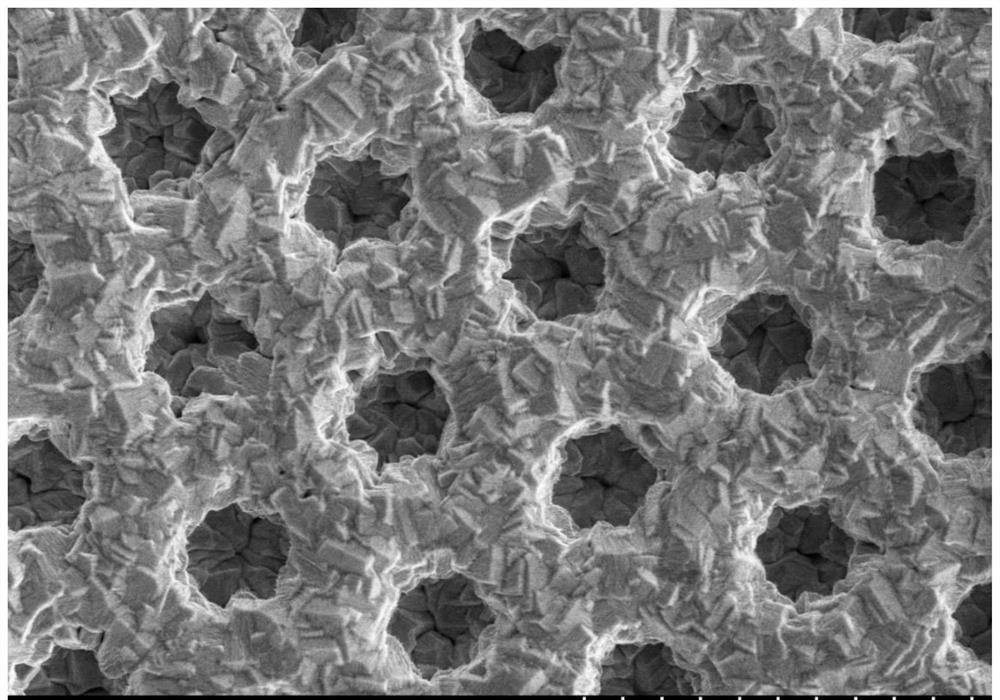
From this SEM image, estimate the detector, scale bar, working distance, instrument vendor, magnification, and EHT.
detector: SE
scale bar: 50000 nm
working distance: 7 mm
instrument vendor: Hitachi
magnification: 1 K X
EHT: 15 kV